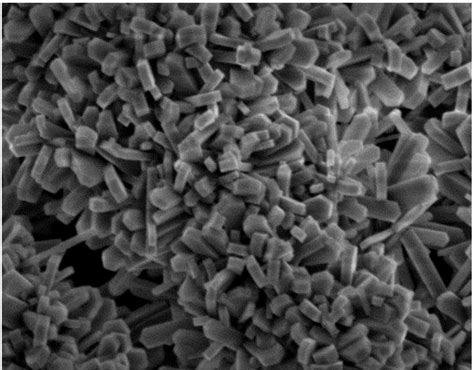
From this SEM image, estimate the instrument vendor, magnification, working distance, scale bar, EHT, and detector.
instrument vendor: JEOL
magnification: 20 K X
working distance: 9.3 mm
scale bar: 1000 nm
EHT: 3 kV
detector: SEI